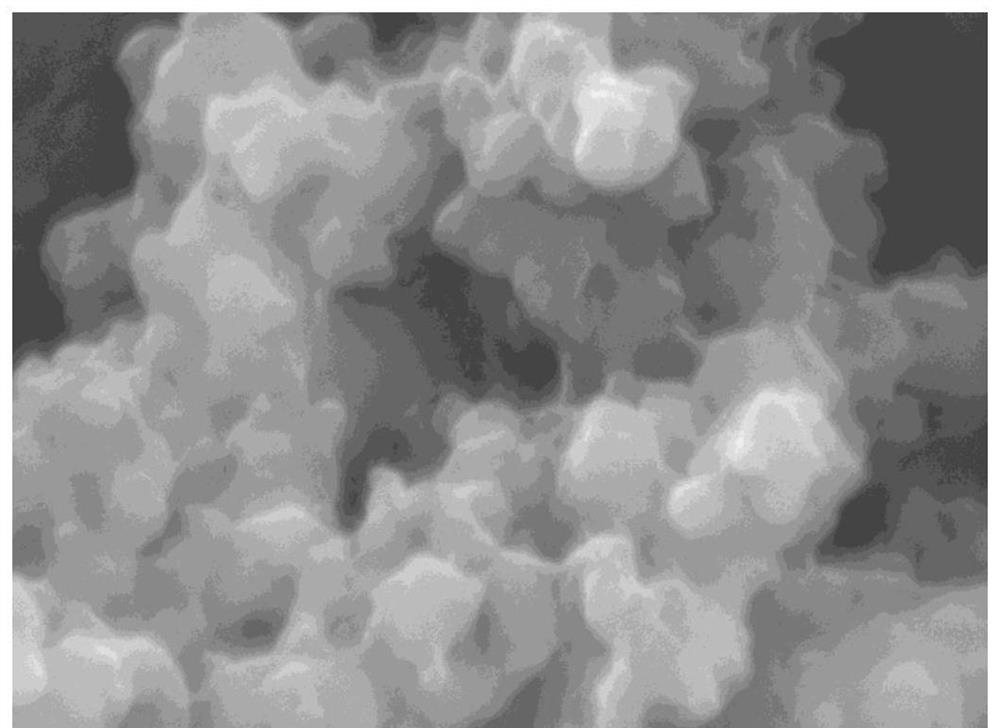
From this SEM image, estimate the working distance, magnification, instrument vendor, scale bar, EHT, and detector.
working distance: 9.6 mm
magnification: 50 K X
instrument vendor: JEOL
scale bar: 100 nm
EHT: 15 kV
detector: LED